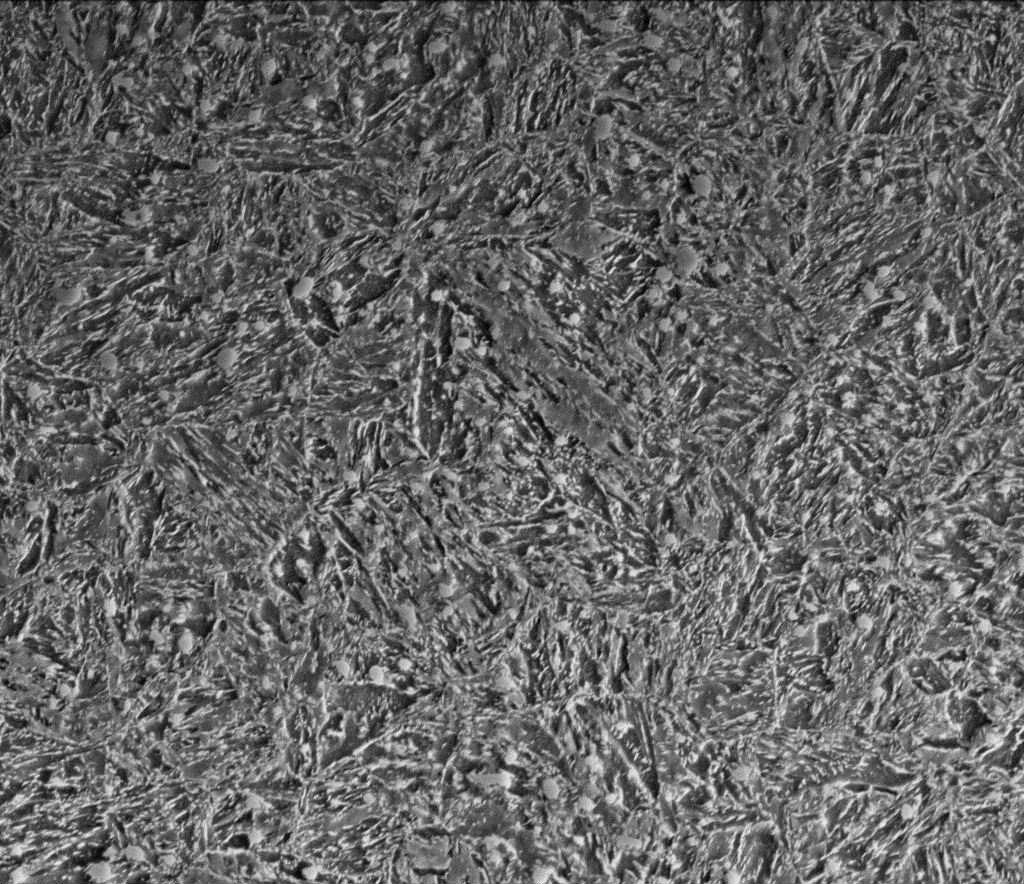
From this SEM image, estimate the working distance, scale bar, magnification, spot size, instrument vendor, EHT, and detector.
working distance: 10 mm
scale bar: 10000 nm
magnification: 10 K X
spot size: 5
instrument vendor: FEI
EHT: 15 kV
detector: ETD